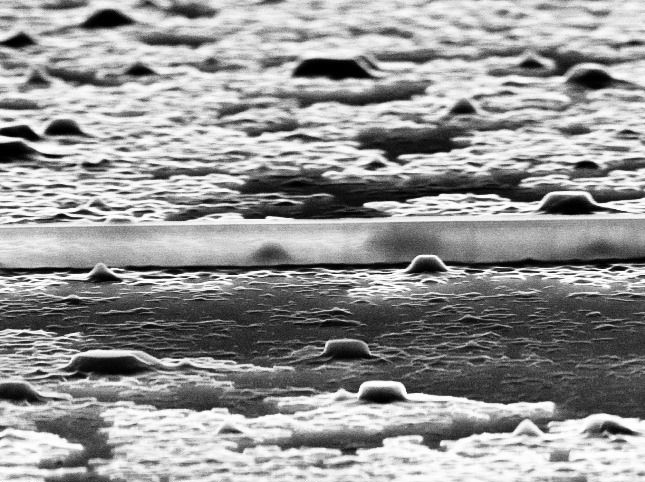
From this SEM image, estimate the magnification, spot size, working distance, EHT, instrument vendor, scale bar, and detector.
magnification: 16.119 K X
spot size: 4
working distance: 15.2 mm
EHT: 30 kV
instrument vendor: FEI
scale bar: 1000 nm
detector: SE